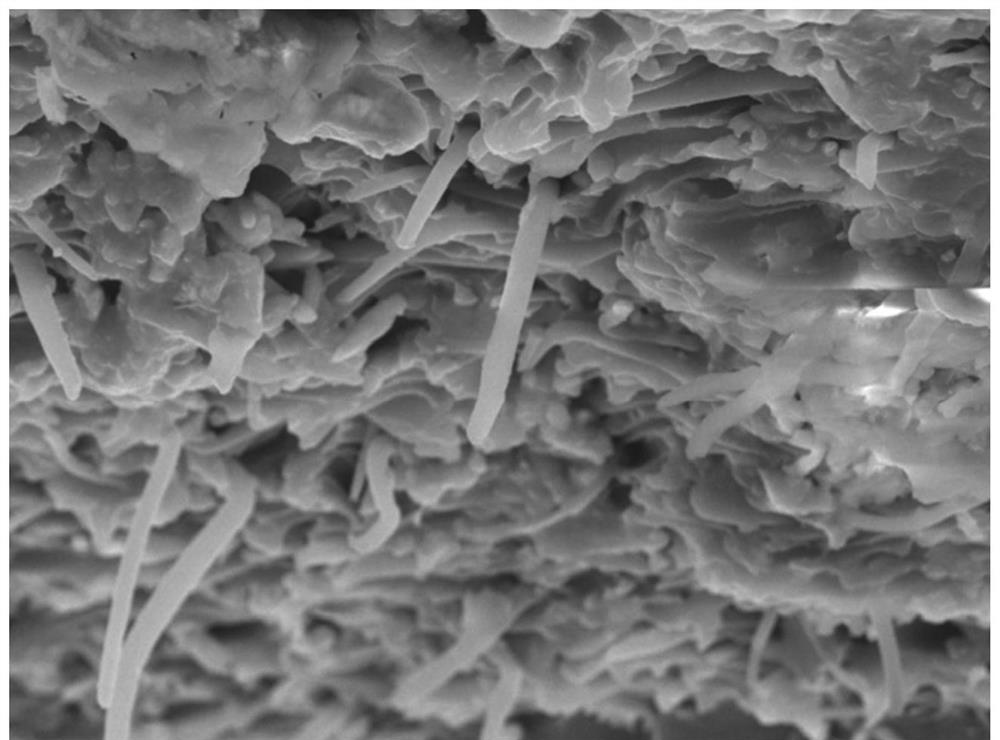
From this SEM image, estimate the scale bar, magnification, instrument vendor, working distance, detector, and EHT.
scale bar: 1000 nm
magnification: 4.3 K X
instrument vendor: JEOL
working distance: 8.8 mm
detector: LED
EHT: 10 kV